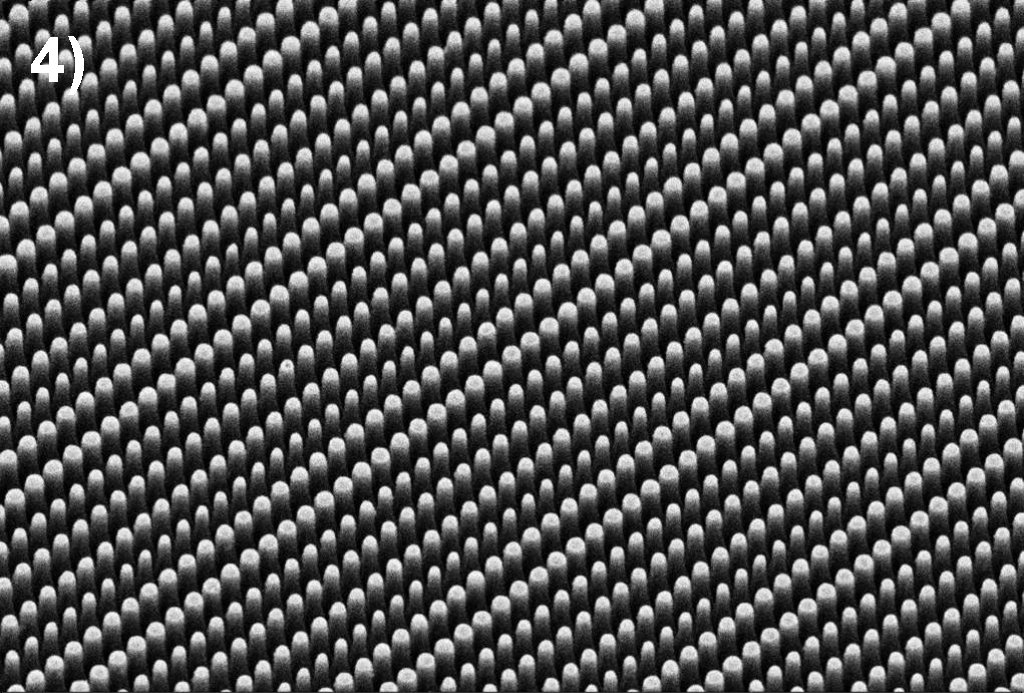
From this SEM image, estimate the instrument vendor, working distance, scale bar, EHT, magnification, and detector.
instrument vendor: Zeiss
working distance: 5.1 mm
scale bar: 1000 nm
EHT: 3 kV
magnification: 11.24 K X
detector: SE2